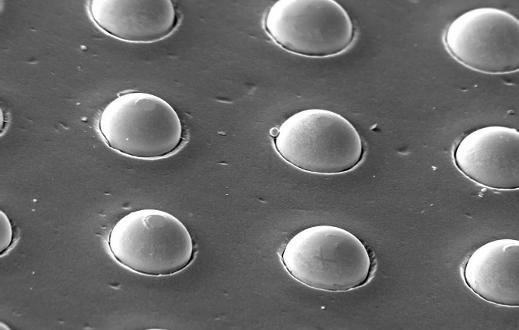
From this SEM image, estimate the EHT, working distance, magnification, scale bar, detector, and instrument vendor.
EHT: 10 kV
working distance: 25 mm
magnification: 0.745 K X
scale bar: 20000 nm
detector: SE1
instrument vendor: Zeiss